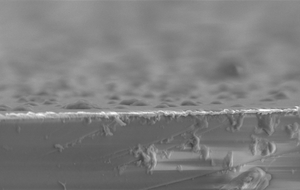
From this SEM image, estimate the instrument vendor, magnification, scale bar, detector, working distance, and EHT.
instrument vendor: Zeiss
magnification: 29.03 K X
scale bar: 1000 nm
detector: SE2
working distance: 13.7 mm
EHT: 3.22 kV